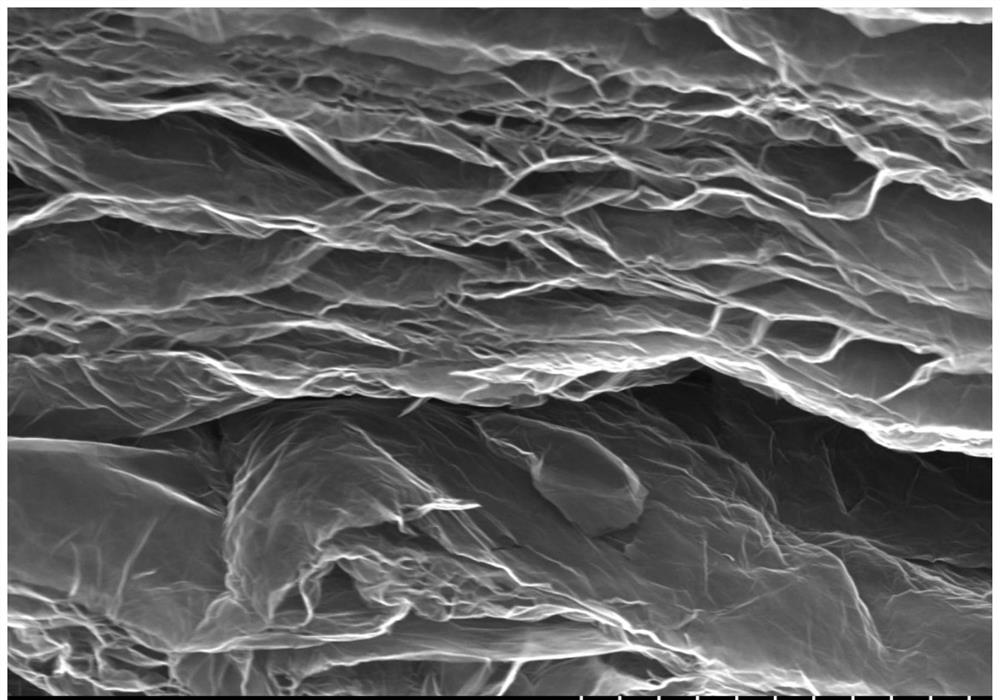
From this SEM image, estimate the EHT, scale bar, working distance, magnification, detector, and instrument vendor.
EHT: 10 kV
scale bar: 5000 nm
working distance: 9.5 mm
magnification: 10 K X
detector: SE(UL)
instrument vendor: Hitachi